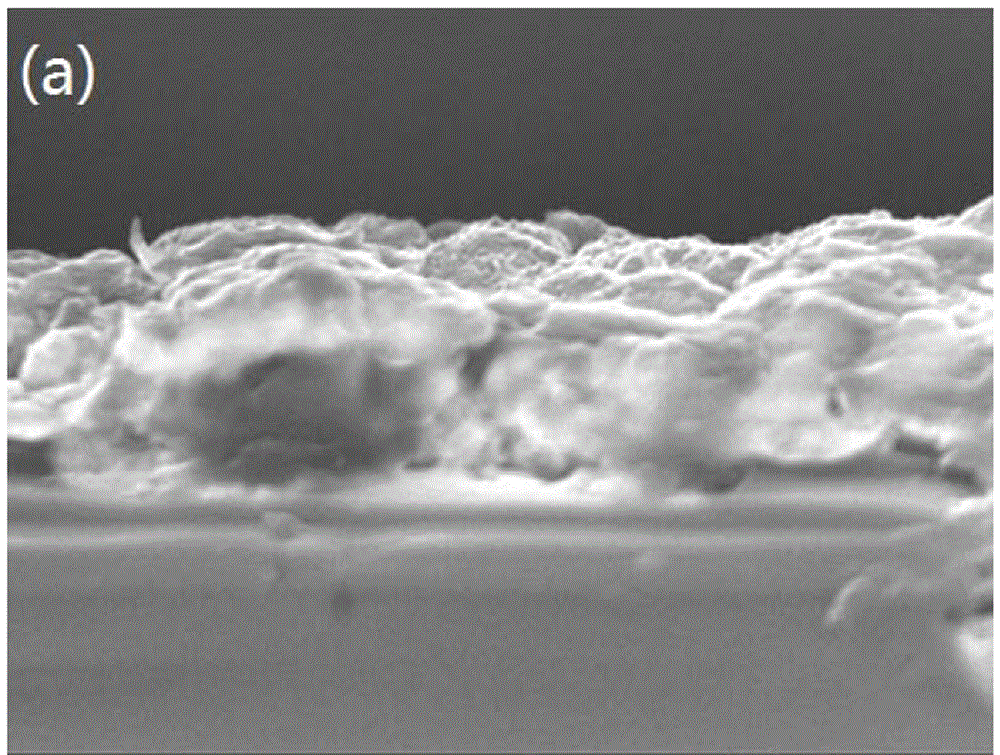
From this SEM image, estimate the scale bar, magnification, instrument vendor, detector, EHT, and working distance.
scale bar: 1000 nm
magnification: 6.5 K X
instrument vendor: JEOL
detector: SEI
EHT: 15 kV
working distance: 3.9 mm